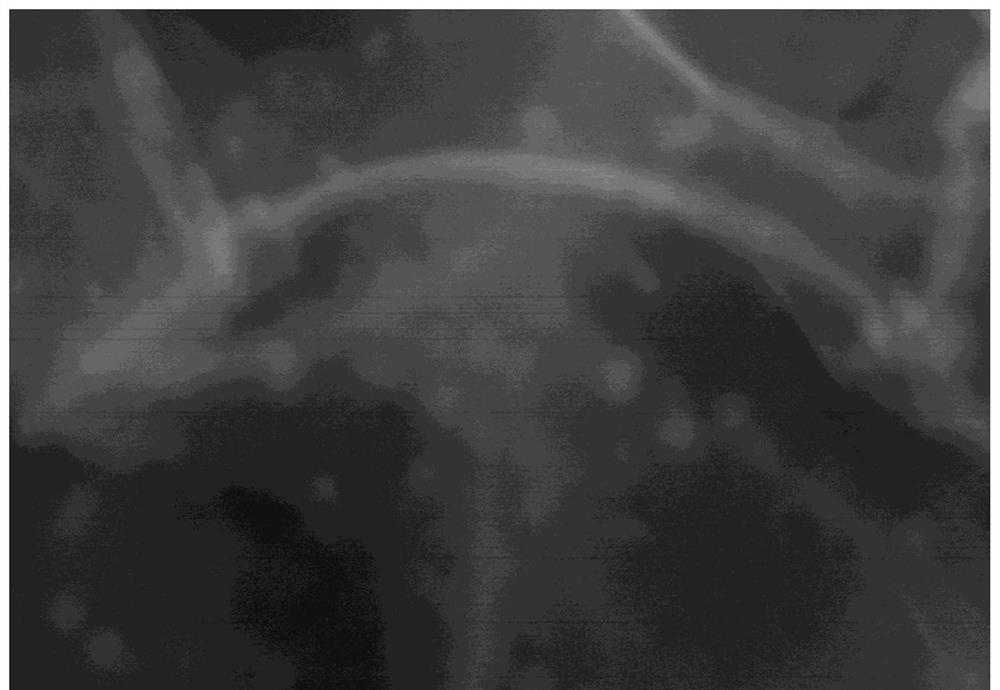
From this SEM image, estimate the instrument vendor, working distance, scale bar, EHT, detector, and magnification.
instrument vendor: Hitachi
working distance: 8.5 mm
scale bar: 100 nm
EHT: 10 kV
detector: SE(M)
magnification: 300 K X